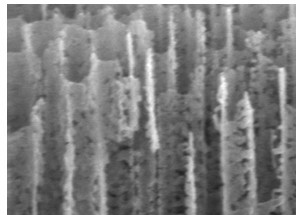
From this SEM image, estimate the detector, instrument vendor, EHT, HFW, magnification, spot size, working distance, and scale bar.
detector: TLD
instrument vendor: FEI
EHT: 5 kV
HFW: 1.2 µm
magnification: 250 K X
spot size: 3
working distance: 4.3 mm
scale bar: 200 nm